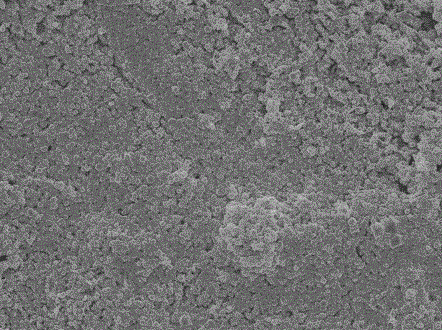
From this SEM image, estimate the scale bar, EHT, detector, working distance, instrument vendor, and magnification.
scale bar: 1000 nm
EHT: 5 kV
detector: SEI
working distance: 7.8 mm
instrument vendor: JEOL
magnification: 6 K X